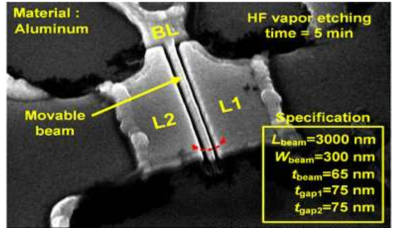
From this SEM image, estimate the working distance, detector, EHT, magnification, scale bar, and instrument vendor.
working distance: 10.6 mm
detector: SE(M)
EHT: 15 kV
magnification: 18 K X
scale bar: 3000 nm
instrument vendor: Hitachi